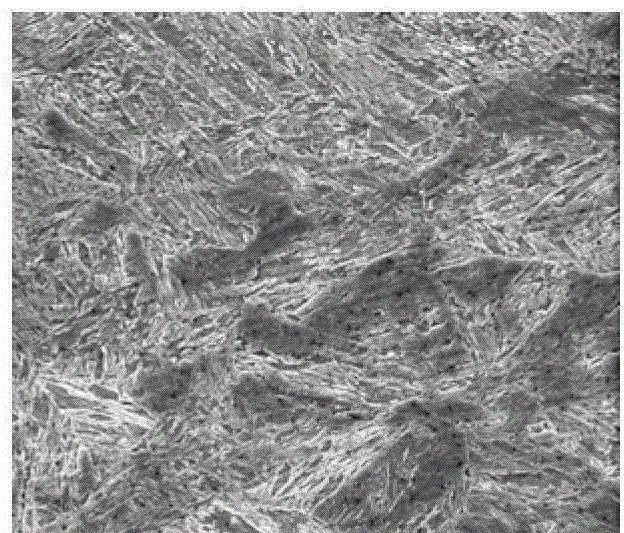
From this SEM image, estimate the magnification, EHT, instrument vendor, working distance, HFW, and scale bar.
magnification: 5 K X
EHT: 20 kV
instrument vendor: FEI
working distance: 14.9 mm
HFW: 59.7 µm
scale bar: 20000 nm